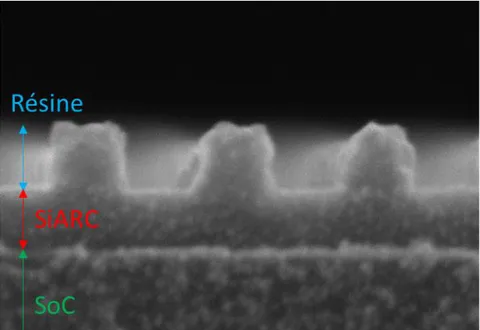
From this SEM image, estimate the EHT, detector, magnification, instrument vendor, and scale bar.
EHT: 5 kV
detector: SE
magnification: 600 K X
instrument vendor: Hitachi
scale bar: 50 nm